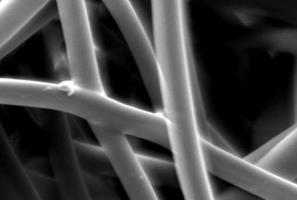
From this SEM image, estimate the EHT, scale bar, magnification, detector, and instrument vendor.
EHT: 10 kV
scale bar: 10000 nm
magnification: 2 K X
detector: SEI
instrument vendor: JEOL